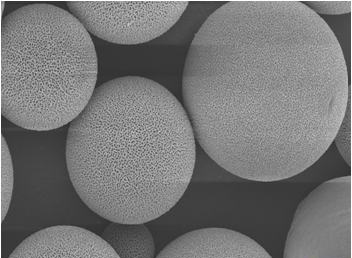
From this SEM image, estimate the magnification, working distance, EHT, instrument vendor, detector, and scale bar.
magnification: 2 K X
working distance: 6.2 mm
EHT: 5 kV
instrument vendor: Zeiss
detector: InLens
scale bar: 2000 nm